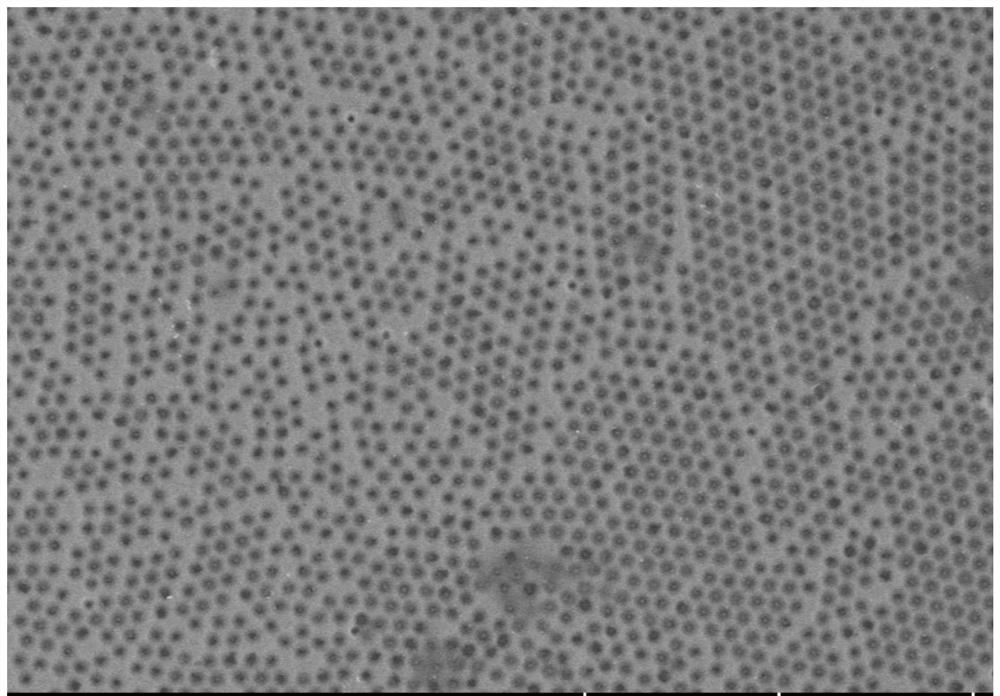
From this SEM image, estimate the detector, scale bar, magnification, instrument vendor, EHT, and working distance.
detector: SE(UL)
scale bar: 10000 nm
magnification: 5 K X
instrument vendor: Hitachi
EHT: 5 kV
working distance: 8.4 mm